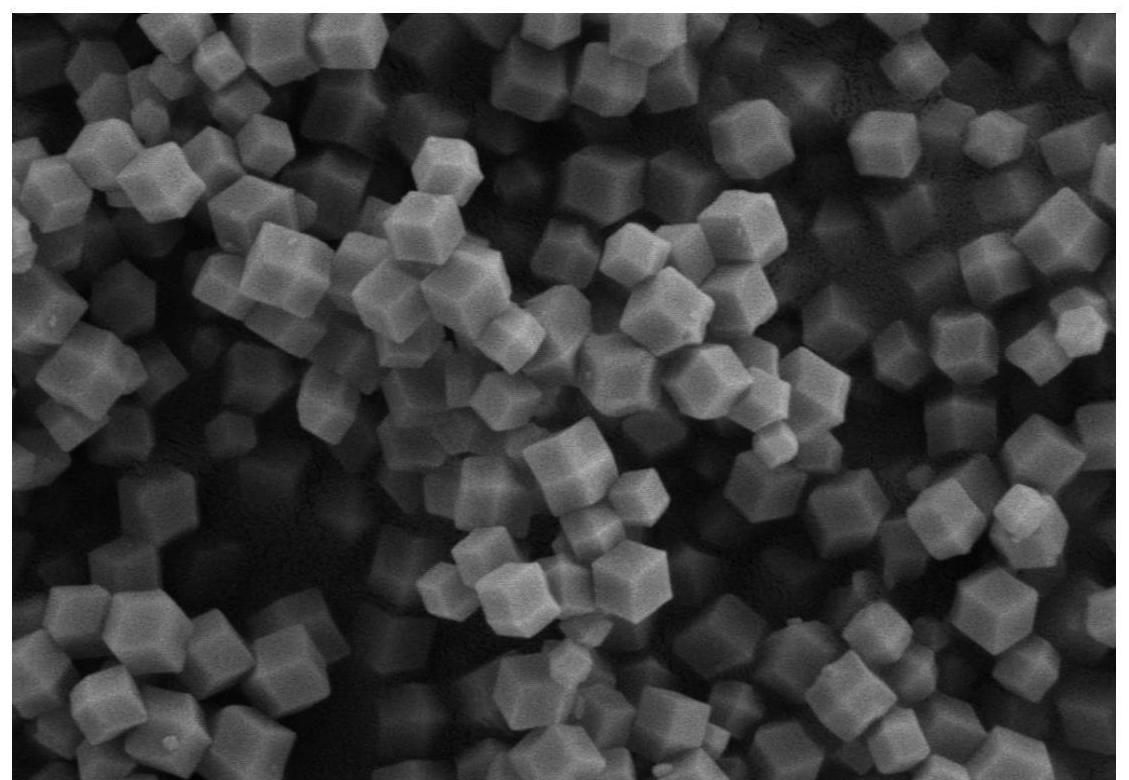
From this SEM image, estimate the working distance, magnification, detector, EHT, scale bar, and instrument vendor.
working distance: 7.2 mm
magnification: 30 K X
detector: SE2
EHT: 3 kV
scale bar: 300 nm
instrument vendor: Zeiss